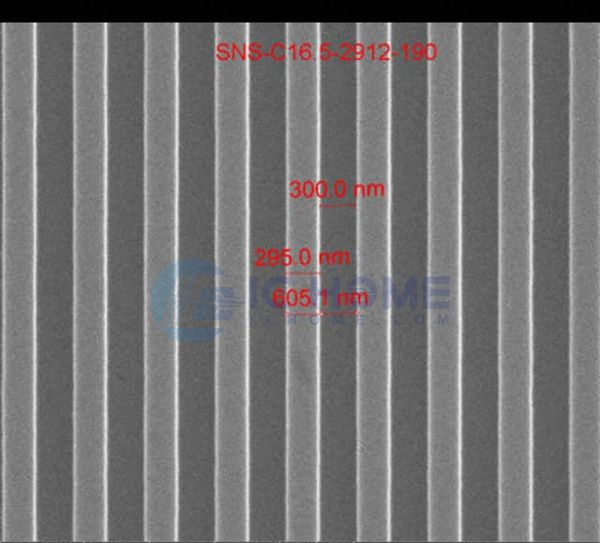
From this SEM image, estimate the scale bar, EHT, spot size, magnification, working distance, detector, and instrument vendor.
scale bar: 2000 nm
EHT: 15 kV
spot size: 1.5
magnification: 50 K X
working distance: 10.7 mm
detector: ETD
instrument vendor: FEI